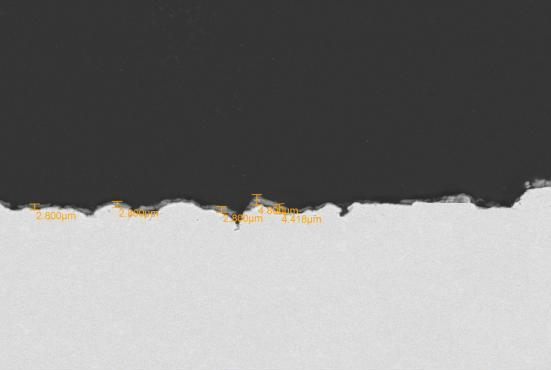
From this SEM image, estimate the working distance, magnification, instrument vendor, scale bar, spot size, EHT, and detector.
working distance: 10 mm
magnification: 0.5 K X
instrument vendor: JEOL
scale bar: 50000 nm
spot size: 40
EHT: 20 kV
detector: BEC